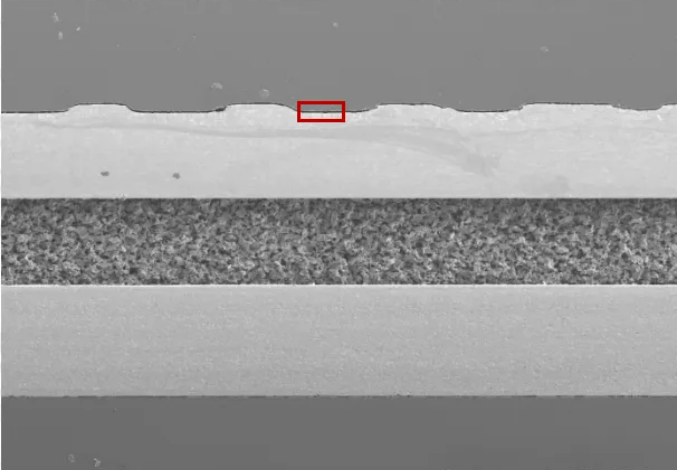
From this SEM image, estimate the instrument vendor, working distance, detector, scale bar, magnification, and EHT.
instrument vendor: Hitachi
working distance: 10.8 mm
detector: LM(L)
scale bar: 1000000 nm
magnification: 0.04 K X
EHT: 10 kV